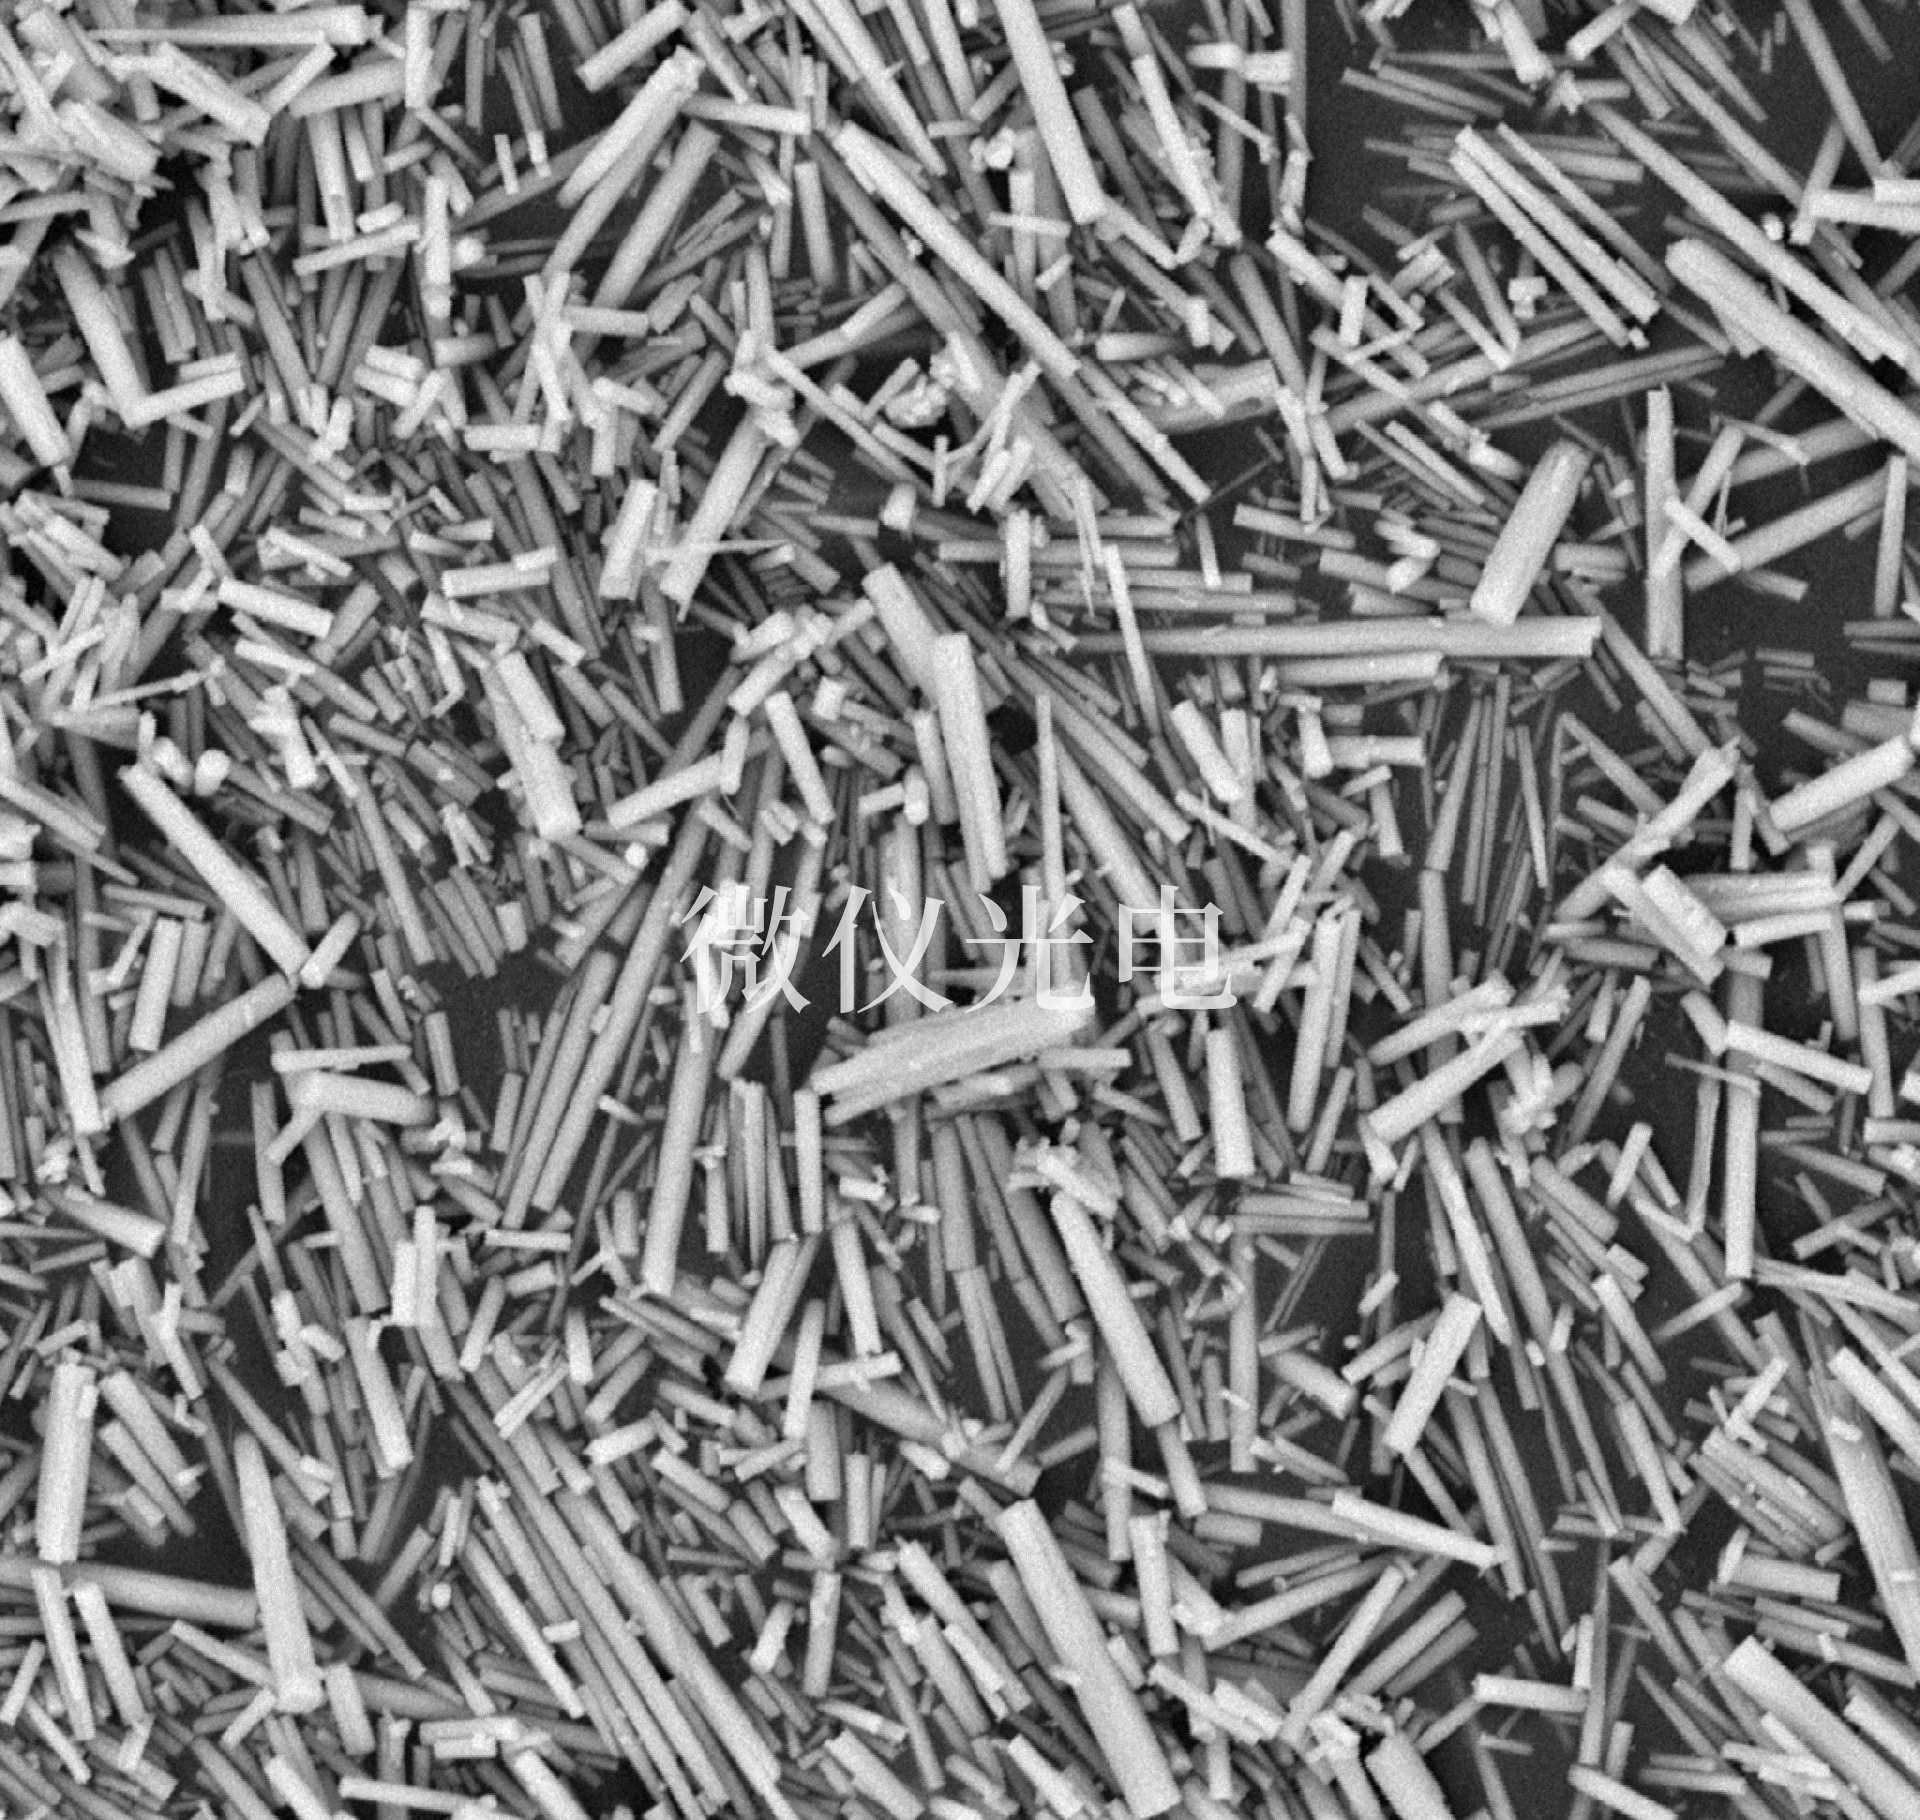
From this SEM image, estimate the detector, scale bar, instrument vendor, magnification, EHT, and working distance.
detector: BSE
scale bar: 5000 nm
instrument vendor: other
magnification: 10 K X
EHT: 15 kV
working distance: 5.8 mm